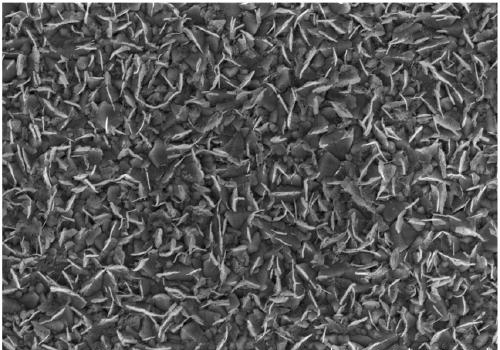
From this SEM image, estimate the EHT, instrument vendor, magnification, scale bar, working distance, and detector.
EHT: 5 kV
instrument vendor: Hitachi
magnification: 20 K X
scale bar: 2000 nm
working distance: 3.6 mm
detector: SE(UL)